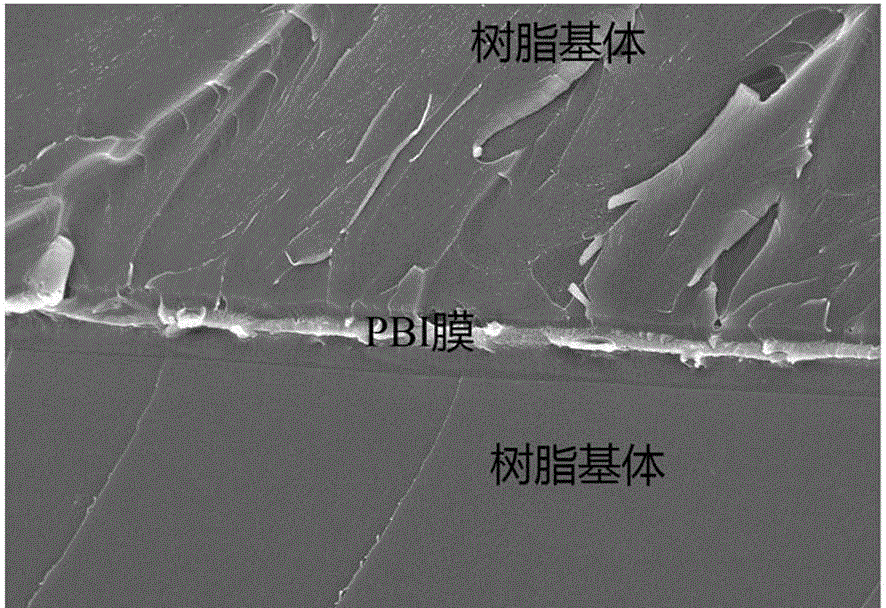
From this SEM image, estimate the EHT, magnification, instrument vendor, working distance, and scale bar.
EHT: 15 kV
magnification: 1 K X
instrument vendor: Hitachi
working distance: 12.8 mm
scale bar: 50000 nm